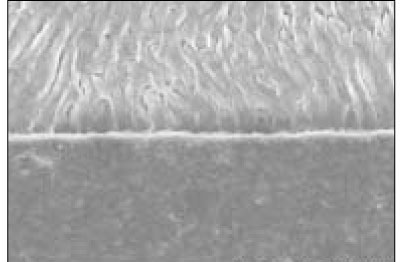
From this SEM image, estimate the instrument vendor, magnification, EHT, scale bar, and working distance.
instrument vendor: Hitachi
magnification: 1 K X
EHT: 15 kV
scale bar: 50000 nm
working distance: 11.8 mm